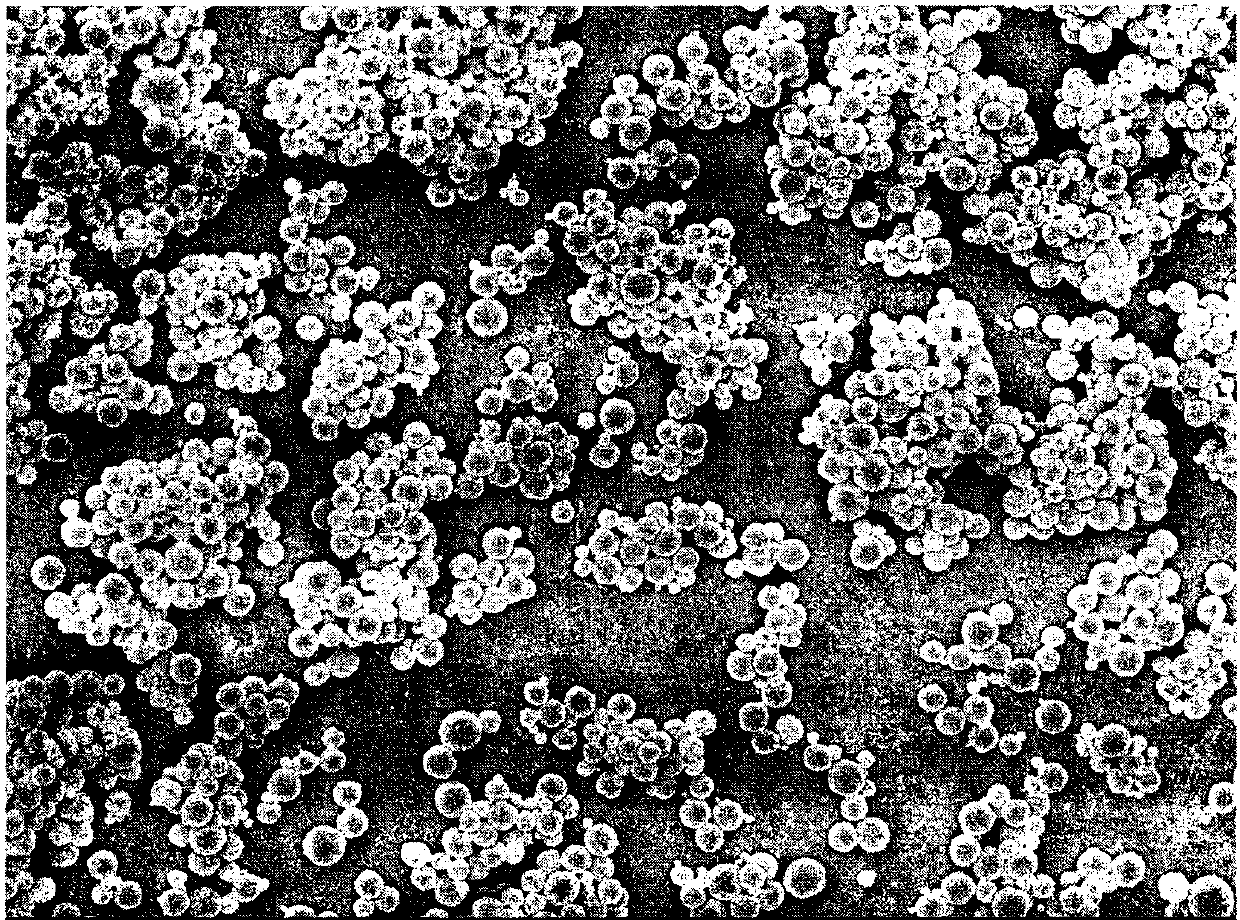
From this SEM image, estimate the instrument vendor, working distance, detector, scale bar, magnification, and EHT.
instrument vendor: JEOL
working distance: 7 mm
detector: SEI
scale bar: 1000 nm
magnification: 5 K X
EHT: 5 kV